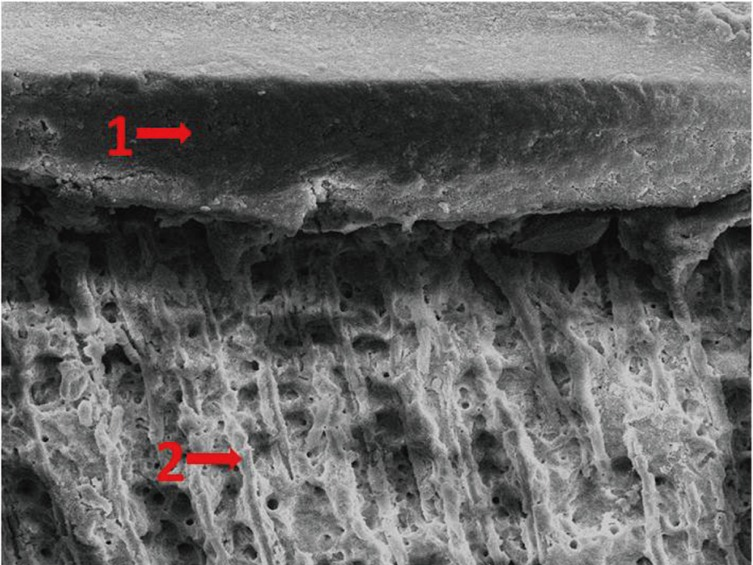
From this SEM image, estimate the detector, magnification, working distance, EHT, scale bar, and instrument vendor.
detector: SEI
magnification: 1 K X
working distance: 10 mm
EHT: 10 kV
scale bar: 10000 nm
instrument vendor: JEOL